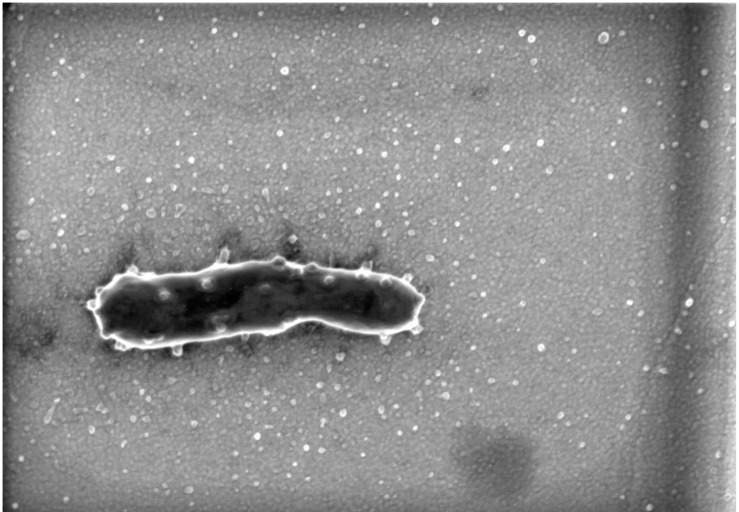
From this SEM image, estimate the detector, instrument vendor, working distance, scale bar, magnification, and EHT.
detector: SE(U,-40)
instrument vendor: Hitachi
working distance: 1.2 mm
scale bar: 1000 nm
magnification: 45 K X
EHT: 0.8 kV